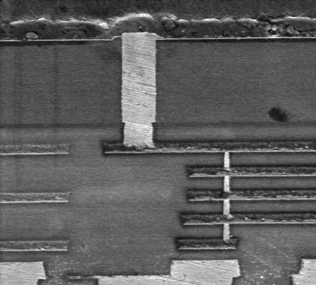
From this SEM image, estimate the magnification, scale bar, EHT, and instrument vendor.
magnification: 5 K X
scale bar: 6000 nm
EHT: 5 kV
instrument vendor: other